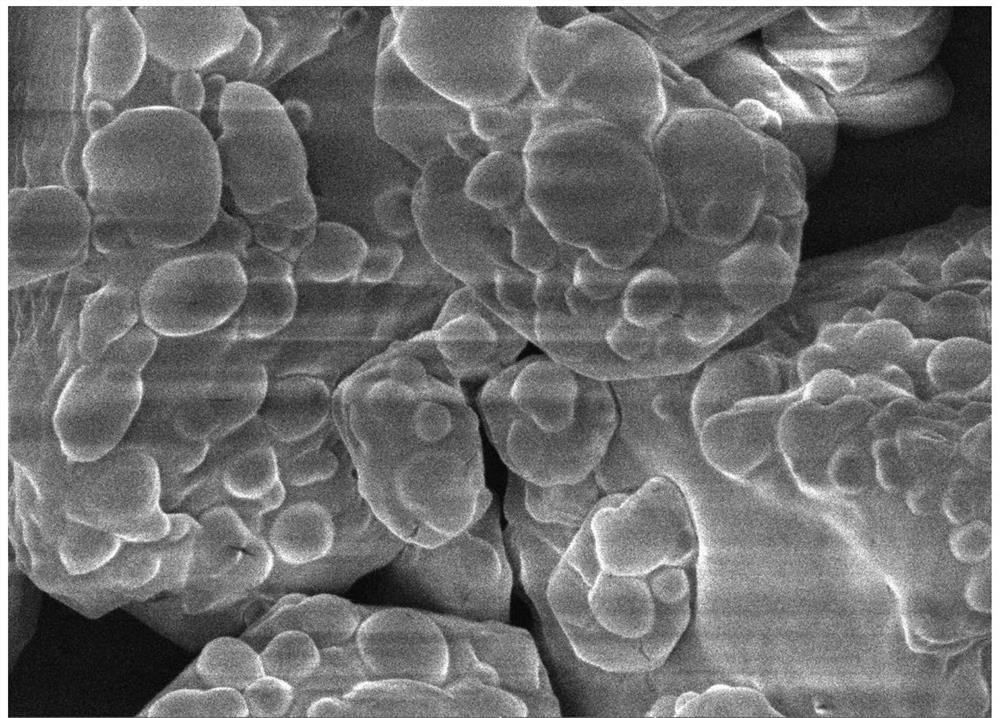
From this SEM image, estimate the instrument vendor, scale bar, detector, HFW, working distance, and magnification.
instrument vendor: Tescan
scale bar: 2000 nm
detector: InBeam SE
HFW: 12.7 µm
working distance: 9.97 mm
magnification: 20 K X